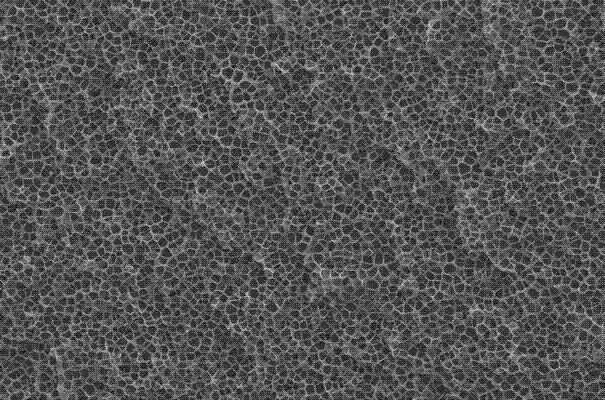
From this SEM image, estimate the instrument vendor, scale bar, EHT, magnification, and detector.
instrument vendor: JEOL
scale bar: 100000 nm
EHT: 20 kV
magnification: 0.2 K X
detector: SEI